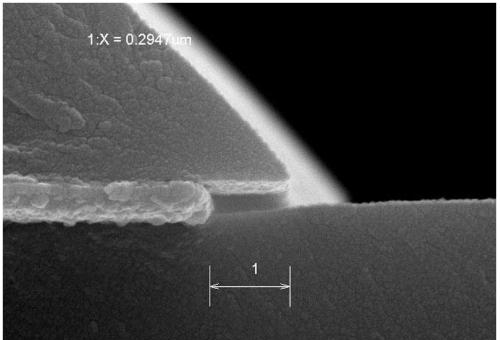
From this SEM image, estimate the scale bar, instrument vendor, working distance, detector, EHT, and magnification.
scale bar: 500 nm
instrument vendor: Hitachi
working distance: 7.4 mm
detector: SE(M)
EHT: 15 kV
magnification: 70 K X